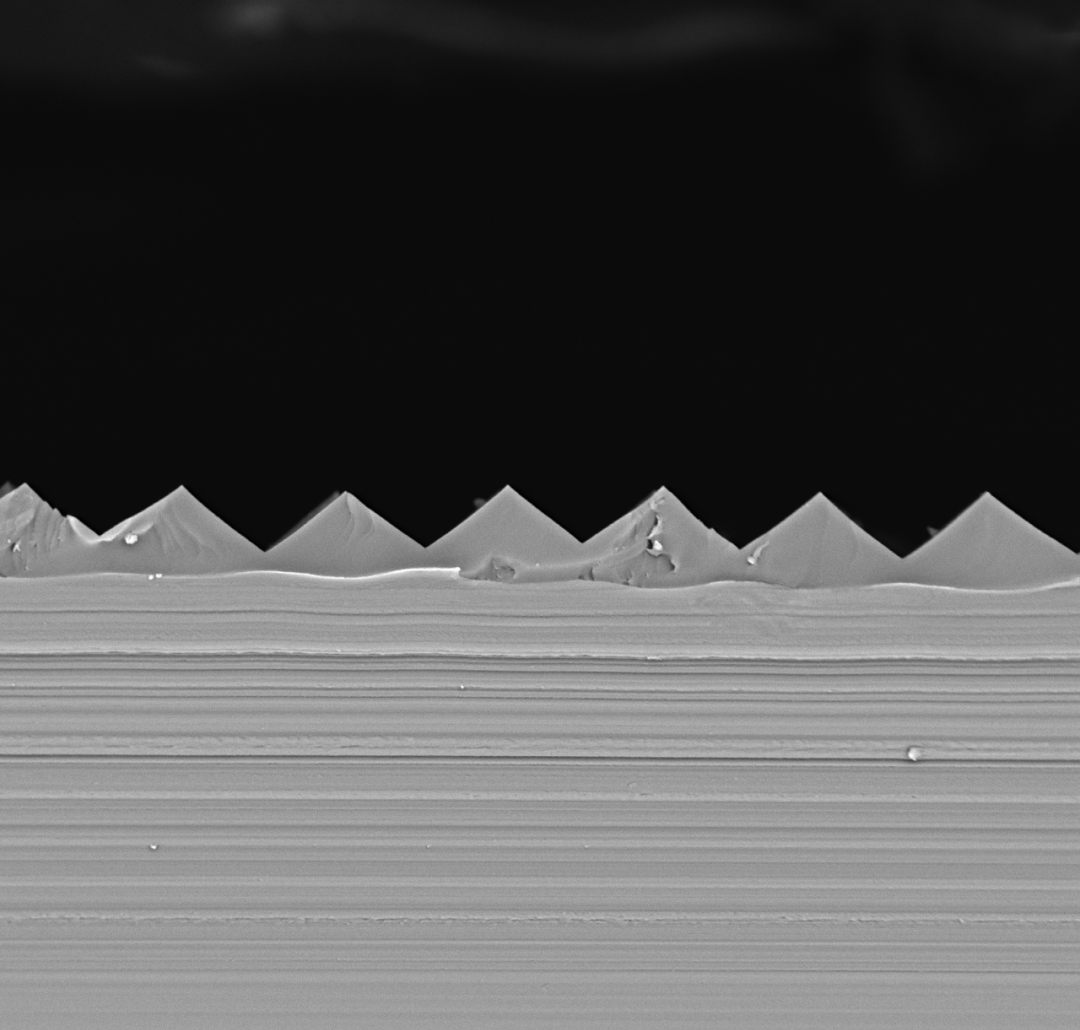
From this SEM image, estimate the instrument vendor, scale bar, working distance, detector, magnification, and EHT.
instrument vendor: other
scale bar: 20000 nm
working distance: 7.1 mm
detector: BSE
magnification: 2 K X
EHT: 10 kV